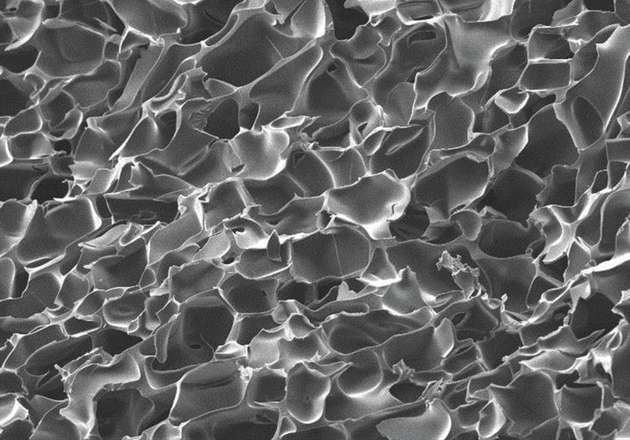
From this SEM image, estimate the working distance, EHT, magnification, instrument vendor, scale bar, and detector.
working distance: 18.3 mm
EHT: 5 kV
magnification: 0.5 K X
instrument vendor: Hitachi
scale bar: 100000 nm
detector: SE(M)